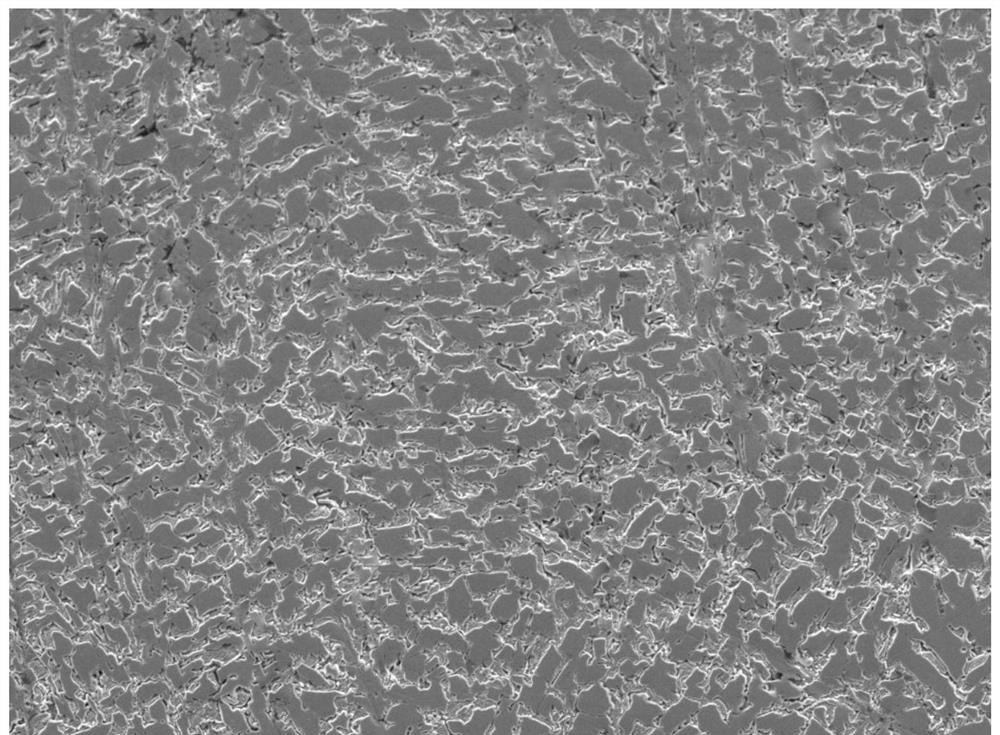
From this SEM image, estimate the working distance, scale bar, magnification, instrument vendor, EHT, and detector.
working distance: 10.1 mm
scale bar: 10000 nm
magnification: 2 K X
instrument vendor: JEOL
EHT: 5 kV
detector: LED